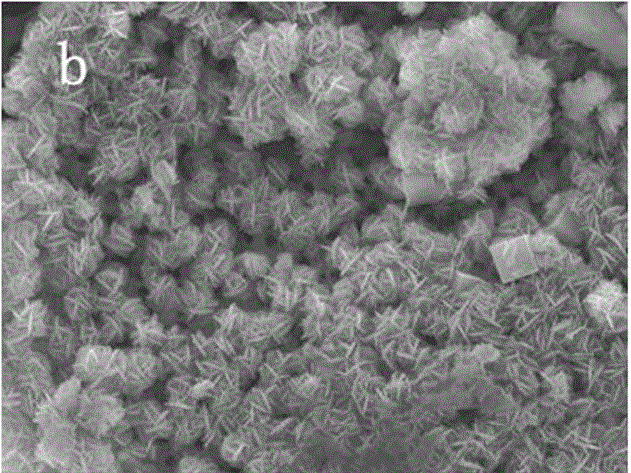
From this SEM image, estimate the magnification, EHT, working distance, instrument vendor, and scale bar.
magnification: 5 K X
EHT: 15 kV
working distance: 20.66 mm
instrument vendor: Tescan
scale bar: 5000 nm